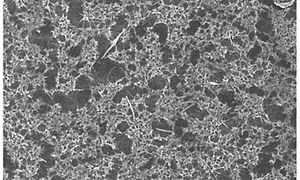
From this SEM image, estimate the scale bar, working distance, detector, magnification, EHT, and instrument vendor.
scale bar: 50000 nm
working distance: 9.6 mm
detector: SE(U)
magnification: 0.8 K X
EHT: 10 kV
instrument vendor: Hitachi